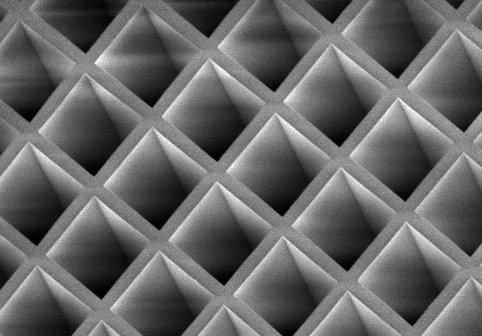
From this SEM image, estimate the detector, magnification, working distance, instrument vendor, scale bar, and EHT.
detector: SE(UL)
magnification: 0.5 K X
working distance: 9.9 mm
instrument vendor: Hitachi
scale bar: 100000 nm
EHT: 2 kV